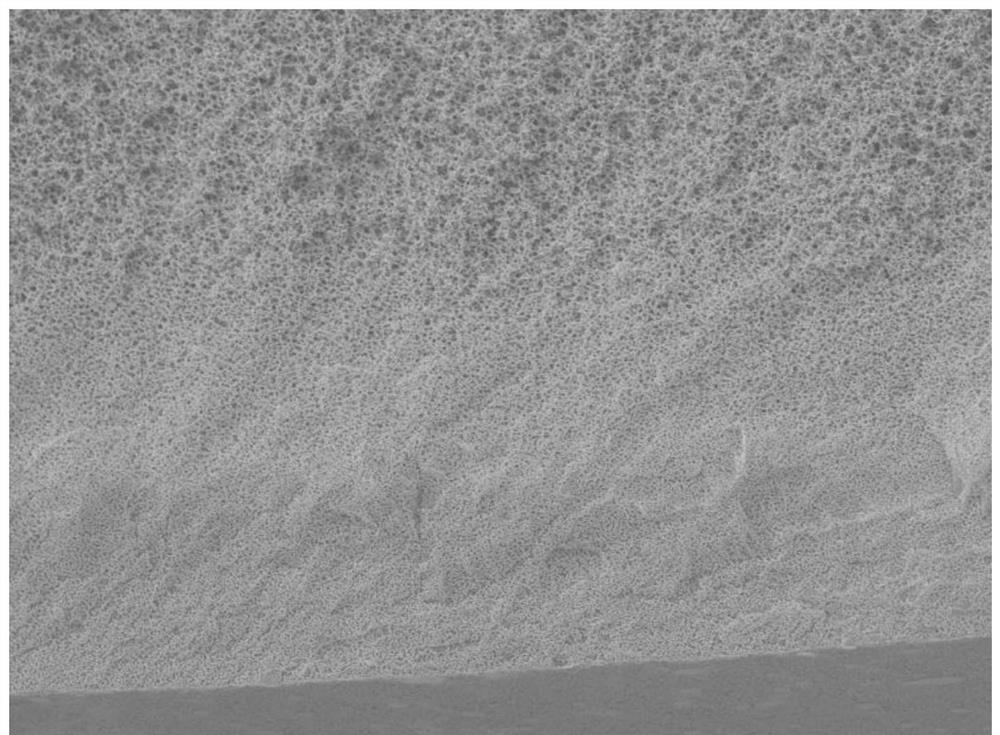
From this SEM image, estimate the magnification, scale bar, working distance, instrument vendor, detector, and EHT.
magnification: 2 K X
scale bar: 10000 nm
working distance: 8.4 mm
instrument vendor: JEOL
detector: LED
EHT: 2 kV